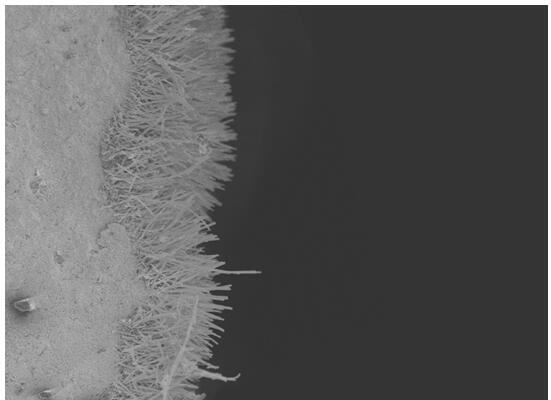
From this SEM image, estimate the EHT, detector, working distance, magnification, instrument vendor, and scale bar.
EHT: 5 kV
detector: SEI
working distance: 8 mm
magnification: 1.5 K X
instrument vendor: JEOL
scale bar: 10000 nm